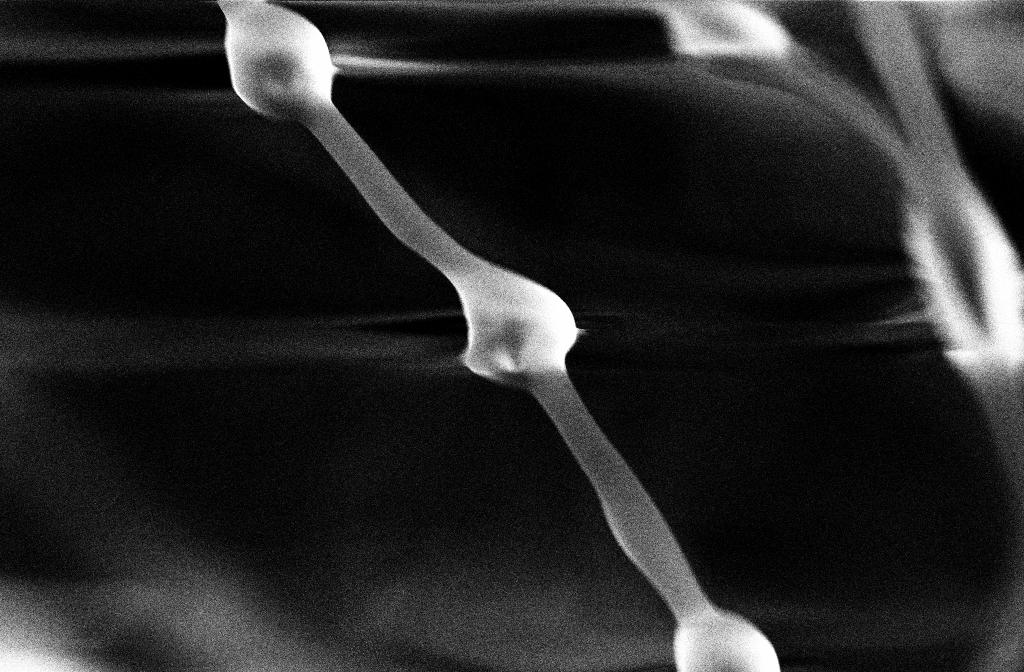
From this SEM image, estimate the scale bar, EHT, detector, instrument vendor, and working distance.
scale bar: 10000 nm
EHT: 20 kV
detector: SE2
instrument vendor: Zeiss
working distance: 18 mm